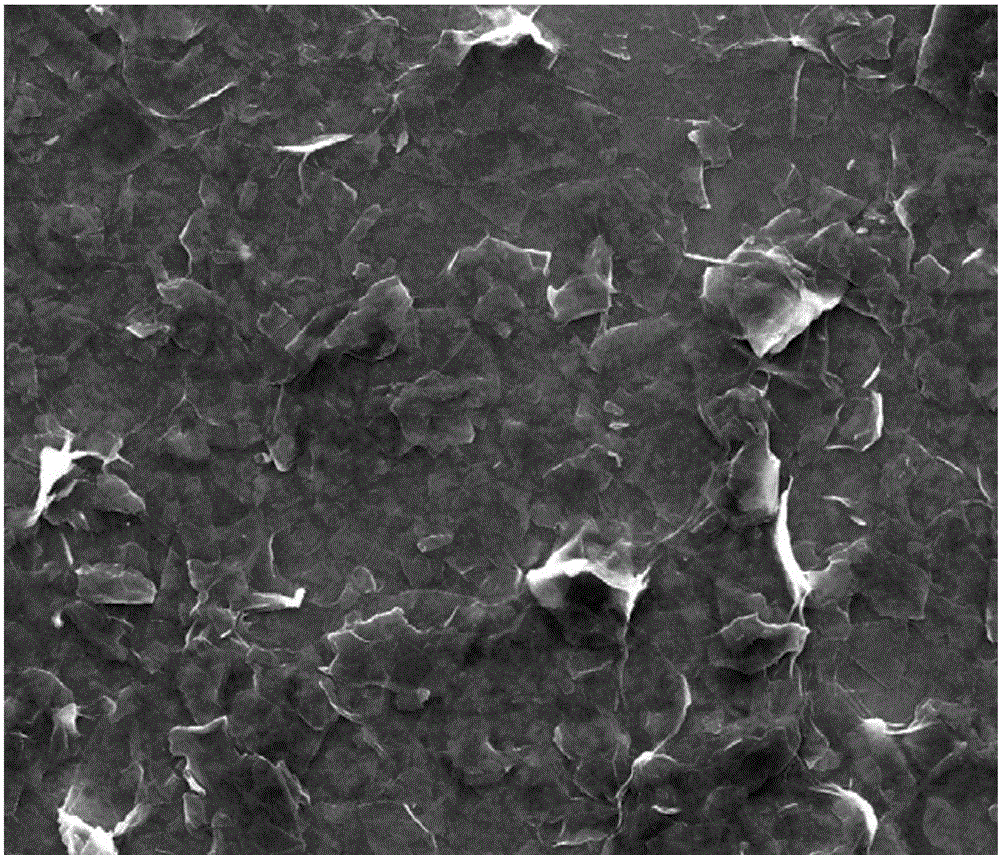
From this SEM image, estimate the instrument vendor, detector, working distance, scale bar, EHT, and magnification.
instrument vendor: FEI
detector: ETD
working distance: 10.5 mm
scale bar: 50000 nm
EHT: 20 kV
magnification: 2.4 K X